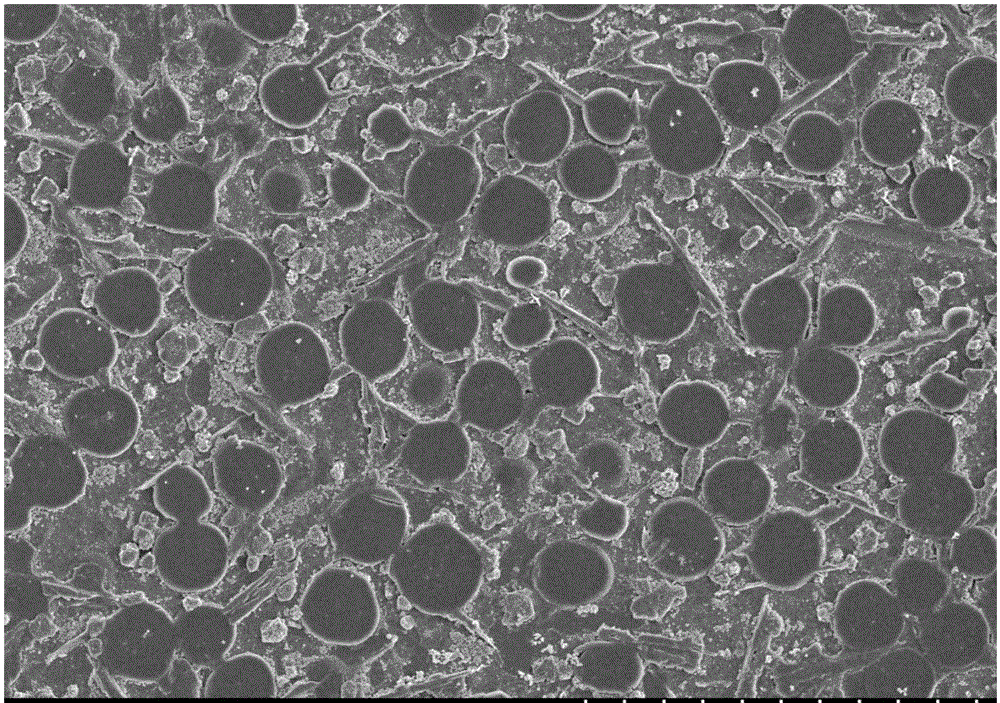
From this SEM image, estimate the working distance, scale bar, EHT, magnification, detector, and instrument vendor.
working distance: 9.8 mm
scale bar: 10000 nm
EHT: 3 kV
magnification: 5 K X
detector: SE(M)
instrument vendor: Hitachi